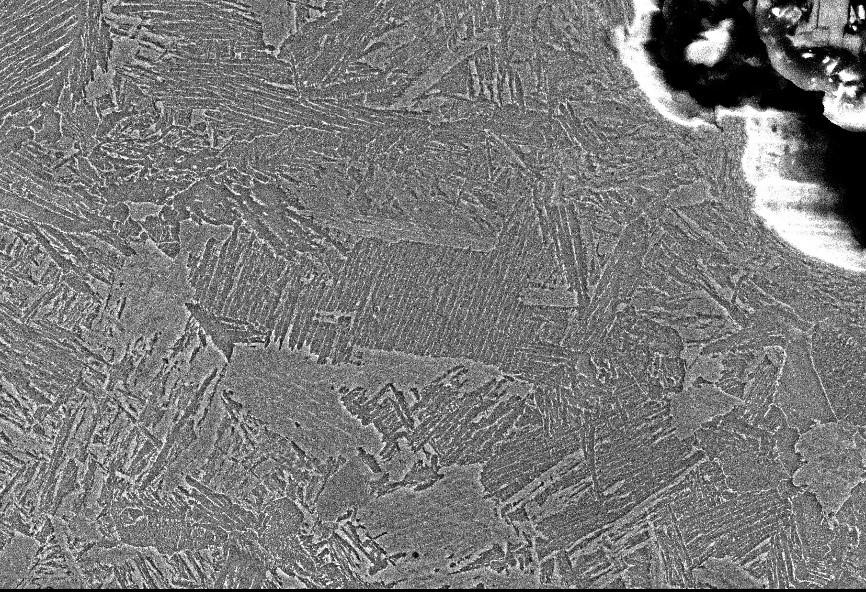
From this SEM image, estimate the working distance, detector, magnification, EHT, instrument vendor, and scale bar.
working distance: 10 mm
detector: SEI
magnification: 1 K X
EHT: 20 kV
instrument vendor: JEOL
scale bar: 10000 nm